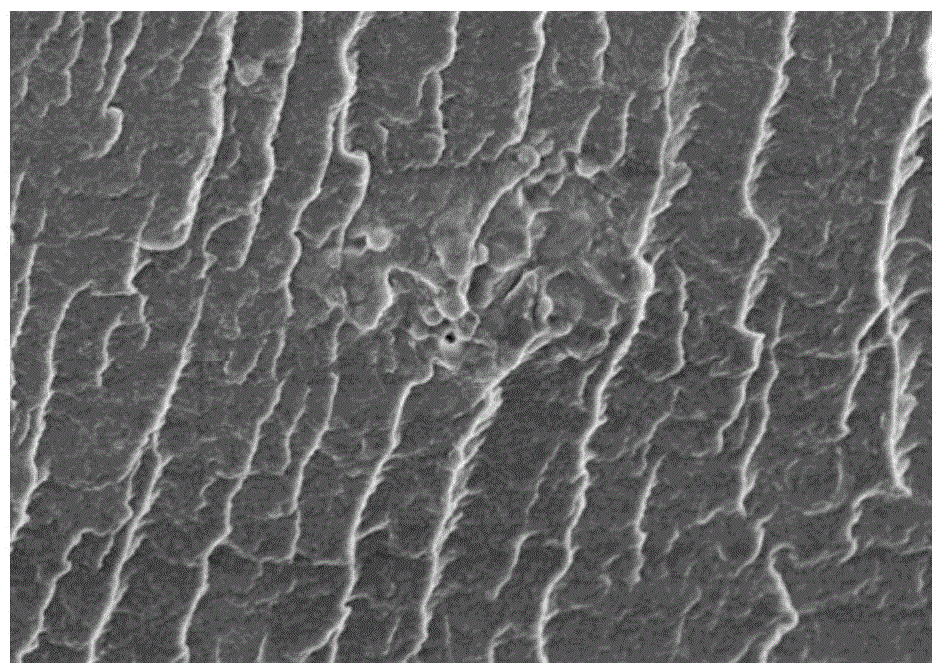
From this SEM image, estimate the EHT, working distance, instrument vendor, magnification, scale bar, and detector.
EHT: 3 kV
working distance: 9 mm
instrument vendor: Hitachi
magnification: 10 K X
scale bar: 5000 nm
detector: SE(M)